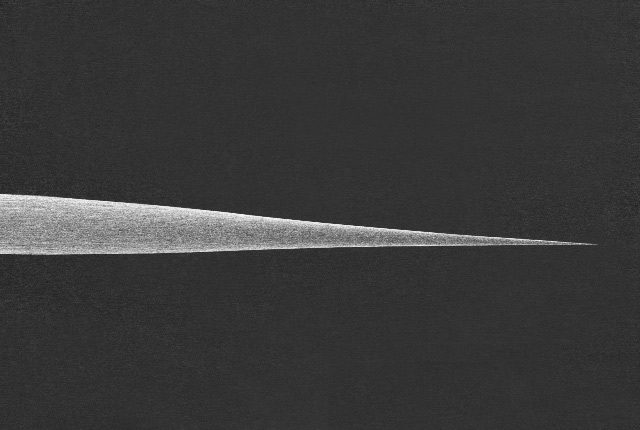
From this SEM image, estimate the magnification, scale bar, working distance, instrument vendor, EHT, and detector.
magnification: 0.04 K X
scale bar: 1000000 nm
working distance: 17.9 mm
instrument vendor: JEOL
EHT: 10 kV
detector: SE